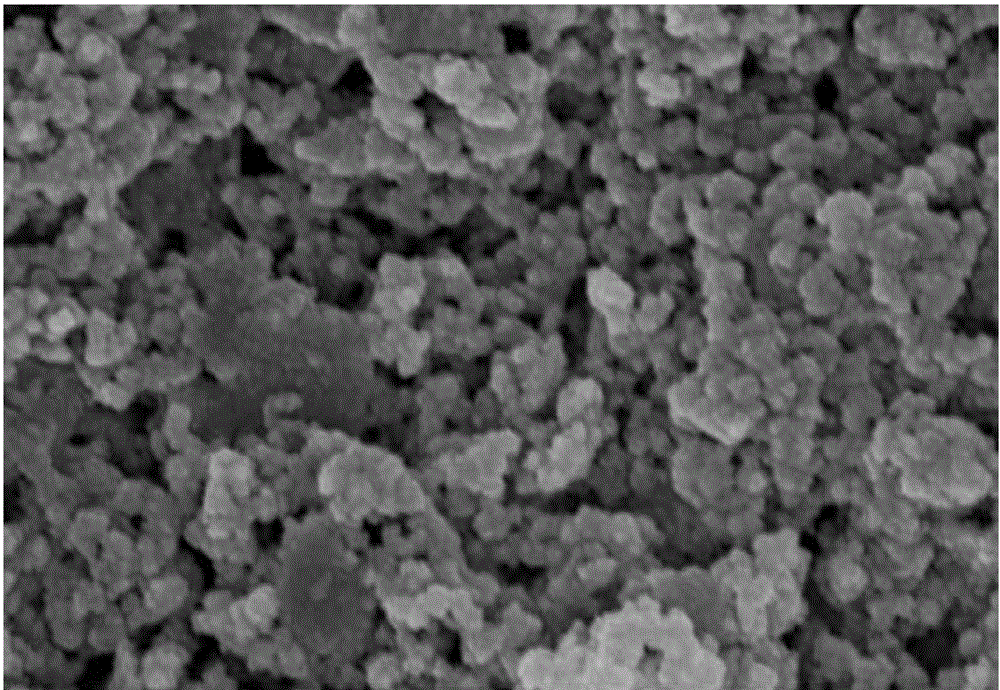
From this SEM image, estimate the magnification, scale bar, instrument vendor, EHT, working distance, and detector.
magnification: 50 K X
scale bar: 100 nm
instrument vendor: Zeiss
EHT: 10 kV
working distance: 7.6 mm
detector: InLens